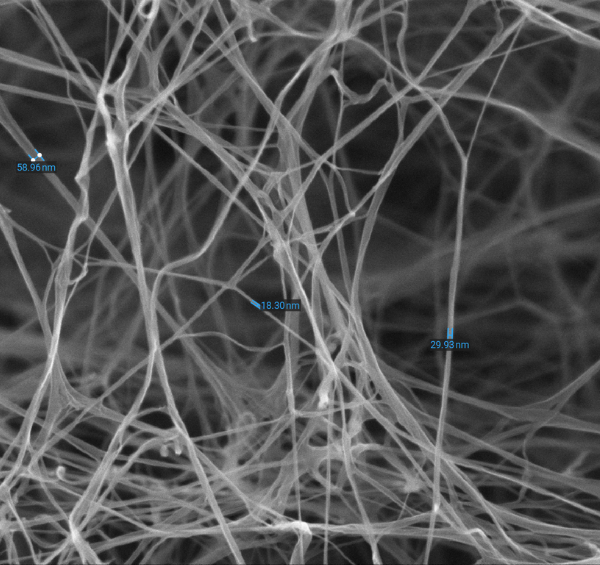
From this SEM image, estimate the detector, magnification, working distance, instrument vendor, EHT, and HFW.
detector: SED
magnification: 20 K X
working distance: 9.057 mm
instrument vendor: Thermo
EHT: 15 kV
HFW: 6.35 µm